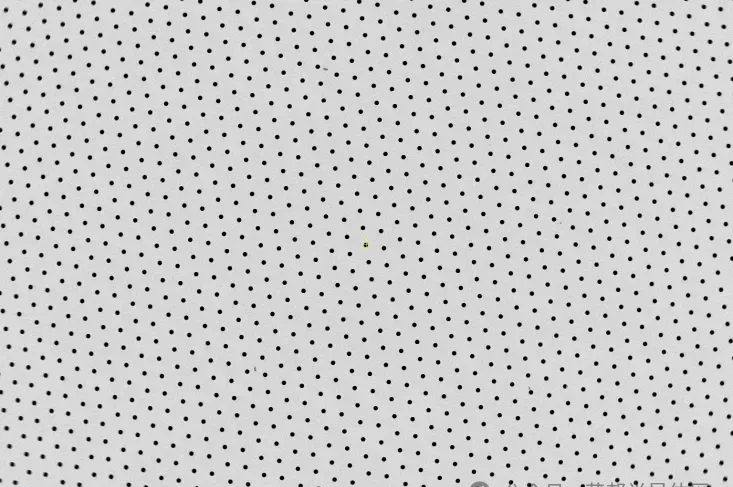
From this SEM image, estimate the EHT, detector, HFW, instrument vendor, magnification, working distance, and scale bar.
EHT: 10 kV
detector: ABS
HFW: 5080 µm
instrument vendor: Thermo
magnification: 0.025 K X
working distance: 10.1 mm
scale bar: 2000000 nm